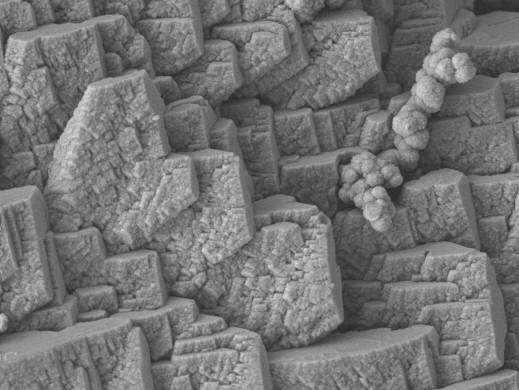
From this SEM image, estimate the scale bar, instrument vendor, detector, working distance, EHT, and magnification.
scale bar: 1000 nm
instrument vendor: JEOL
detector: LEI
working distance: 8.1 mm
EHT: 5 kV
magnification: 20 K X